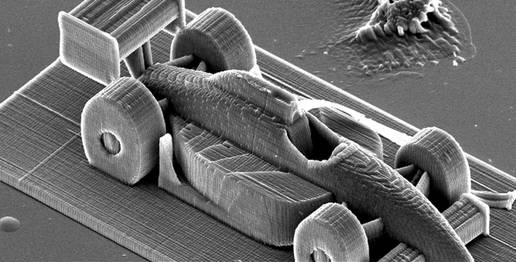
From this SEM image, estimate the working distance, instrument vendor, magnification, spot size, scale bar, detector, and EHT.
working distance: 27.2 mm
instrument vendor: FEI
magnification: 0.364 K X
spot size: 4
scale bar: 100000 nm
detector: SE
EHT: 20 kV